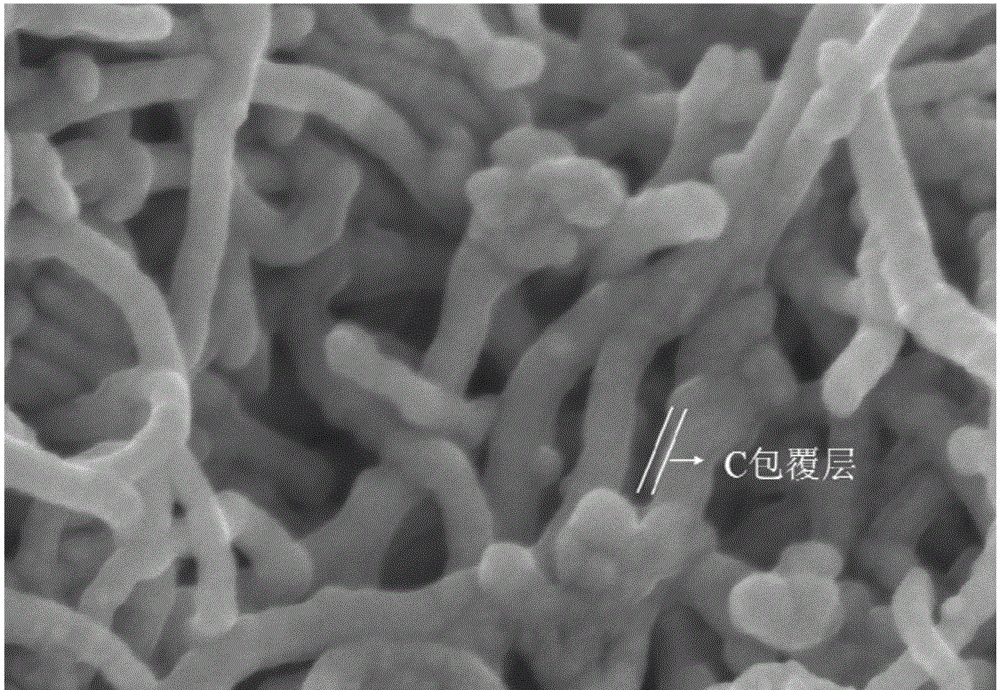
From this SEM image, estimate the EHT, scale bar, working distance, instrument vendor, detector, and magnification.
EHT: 3 kV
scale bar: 500 nm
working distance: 6.3 mm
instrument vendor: Hitachi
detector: SE(U)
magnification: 100 K X